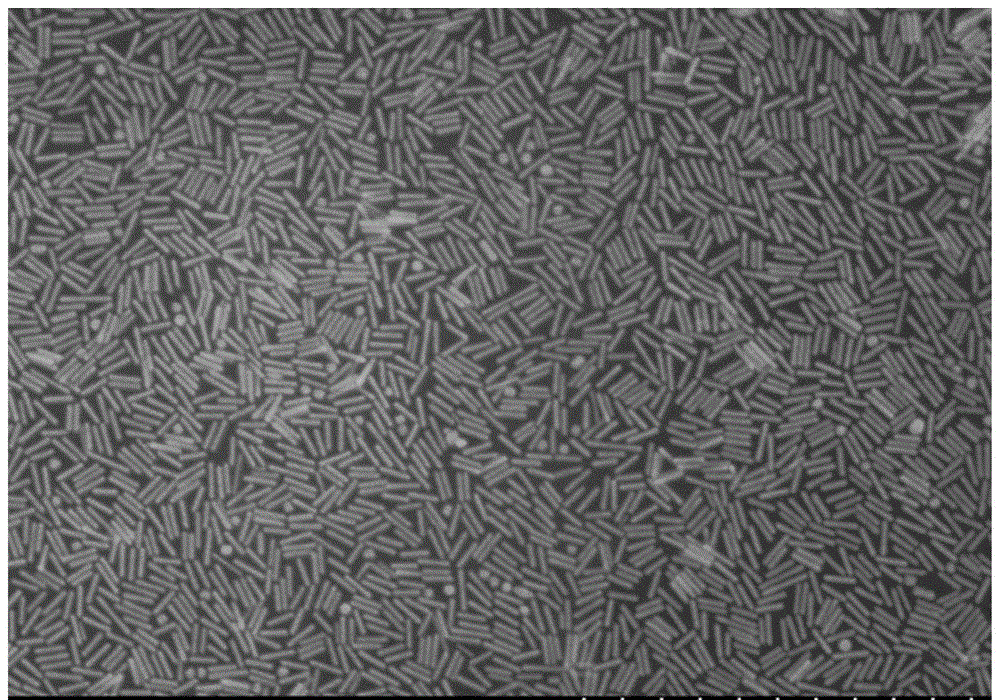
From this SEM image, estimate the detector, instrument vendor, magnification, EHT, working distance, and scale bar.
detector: SE(M)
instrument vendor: Hitachi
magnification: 50 K X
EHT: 5 kV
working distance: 9.5 mm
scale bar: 1000 nm